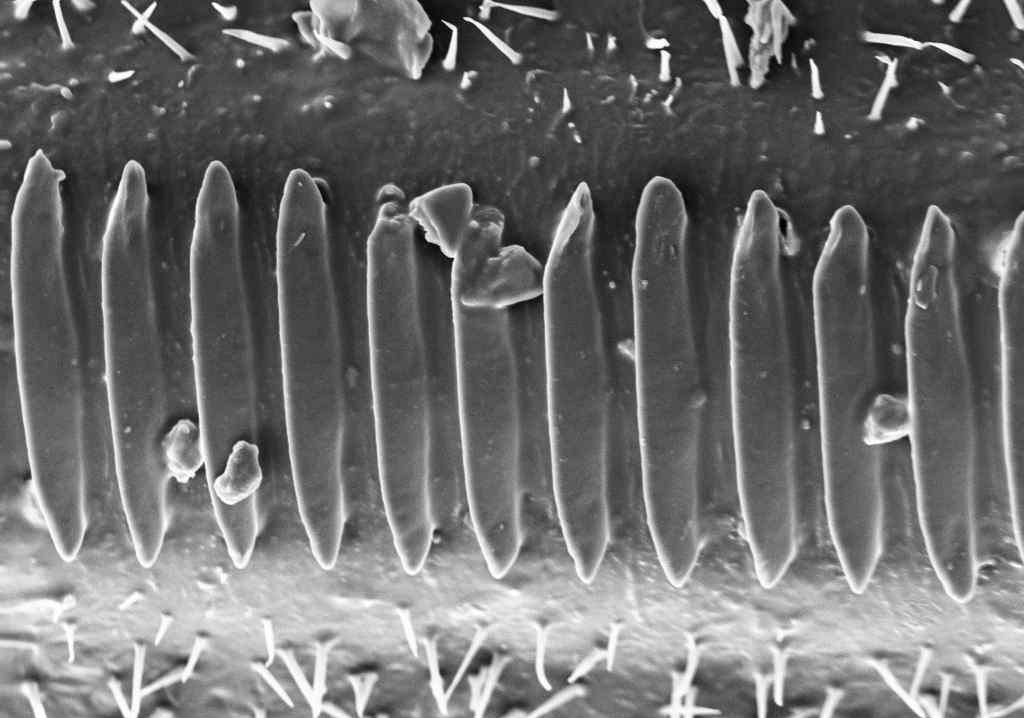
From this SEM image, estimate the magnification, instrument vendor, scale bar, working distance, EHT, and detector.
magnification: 2 K X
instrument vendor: Zeiss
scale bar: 10000 nm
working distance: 6 mm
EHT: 10 kV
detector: SE1